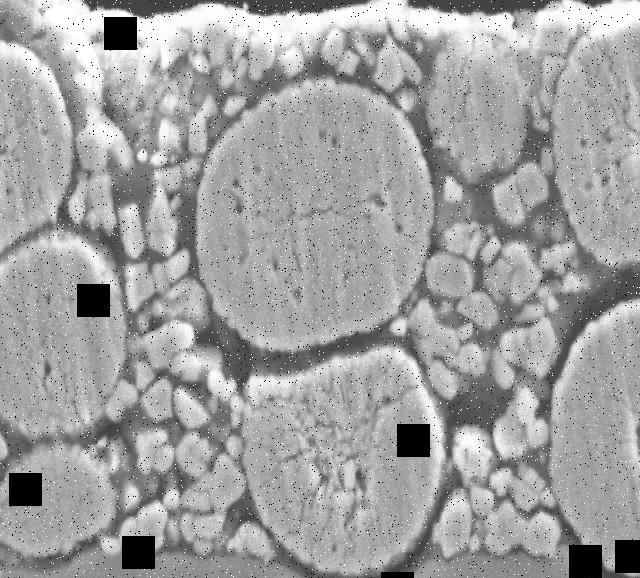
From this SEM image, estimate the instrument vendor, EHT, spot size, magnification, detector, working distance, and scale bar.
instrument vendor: JEOL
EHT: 15 kV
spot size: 60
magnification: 3 K X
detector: SEI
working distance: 8 mm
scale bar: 5000 nm